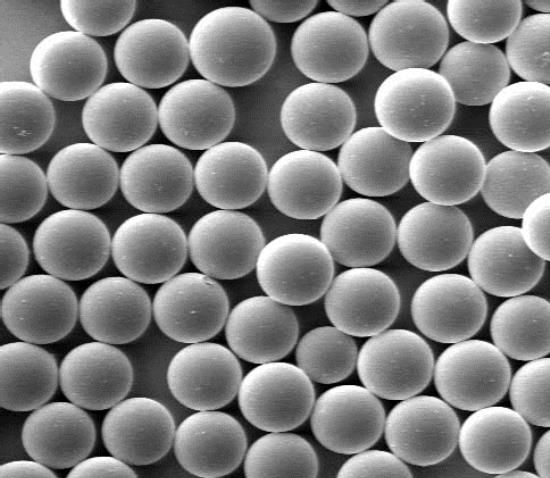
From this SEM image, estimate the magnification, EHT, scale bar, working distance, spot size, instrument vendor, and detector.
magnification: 1.5 K X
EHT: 3 kV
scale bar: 20000 nm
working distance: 4.9 mm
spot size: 3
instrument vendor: FEI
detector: SE